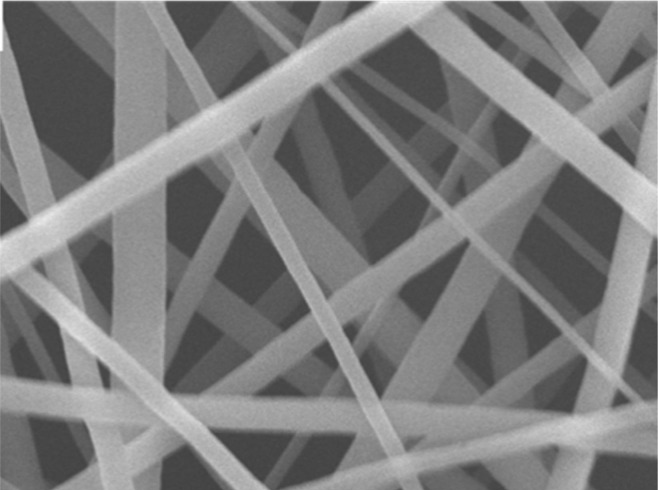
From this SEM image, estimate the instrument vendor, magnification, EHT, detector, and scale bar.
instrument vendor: JEOL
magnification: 10 K X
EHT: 20 kV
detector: SEI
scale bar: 1000 nm